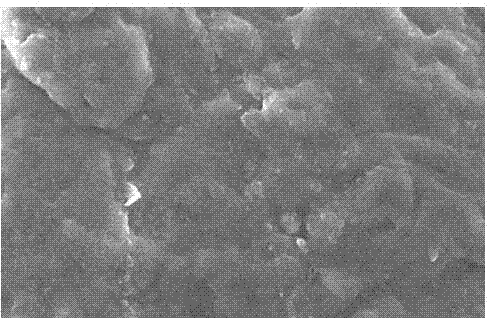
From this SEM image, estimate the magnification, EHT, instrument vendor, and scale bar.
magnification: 4 K X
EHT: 10 kV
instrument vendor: JEOL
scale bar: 5000 nm